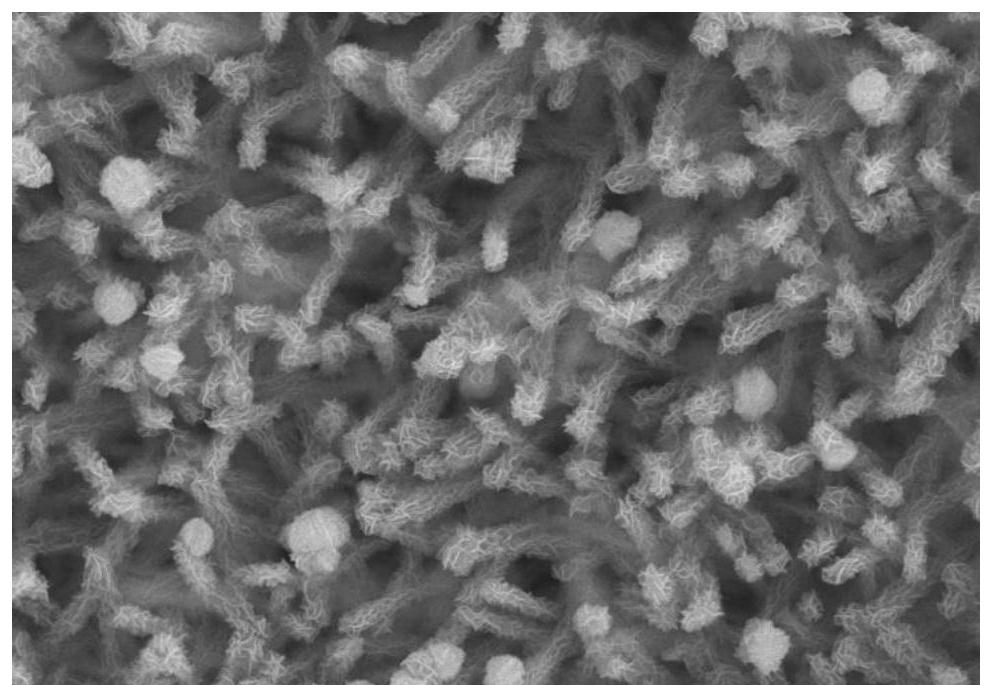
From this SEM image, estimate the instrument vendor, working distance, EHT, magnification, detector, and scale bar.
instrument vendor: Hitachi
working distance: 11 mm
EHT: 15 kV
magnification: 10 K X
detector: SE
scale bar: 5000 nm